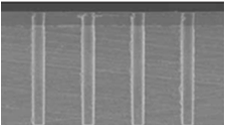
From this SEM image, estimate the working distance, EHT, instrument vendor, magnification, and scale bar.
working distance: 12 mm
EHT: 5 kV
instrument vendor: Hitachi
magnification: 1.1 K X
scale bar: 50000 nm